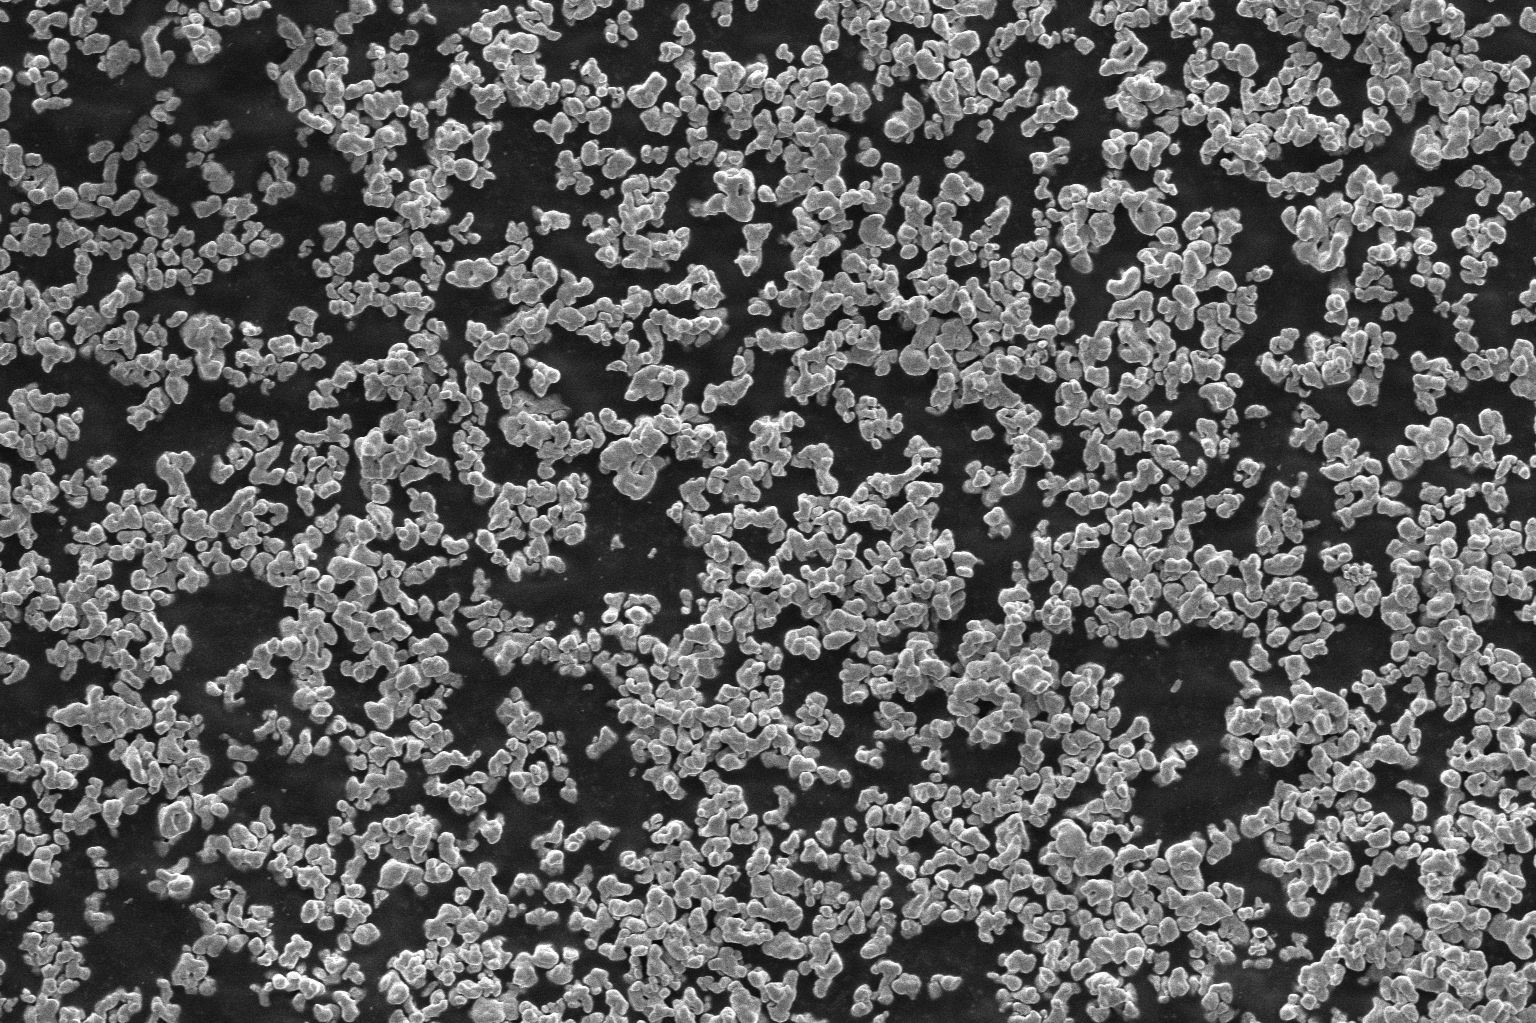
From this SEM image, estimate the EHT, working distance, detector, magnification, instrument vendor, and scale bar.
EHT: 5 kV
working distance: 9.7 mm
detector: ETD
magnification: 3 K X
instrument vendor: FEI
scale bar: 30000 nm